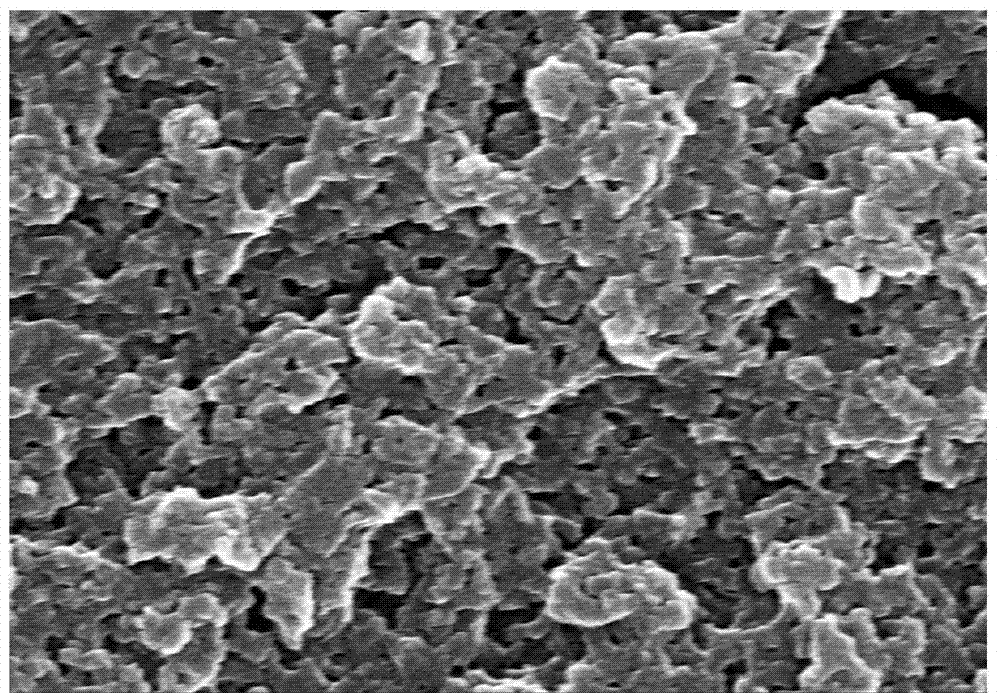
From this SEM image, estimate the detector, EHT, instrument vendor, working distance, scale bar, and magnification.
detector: SE(M)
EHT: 20 kV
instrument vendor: Hitachi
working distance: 15.9 mm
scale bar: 2000 nm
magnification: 20 K X